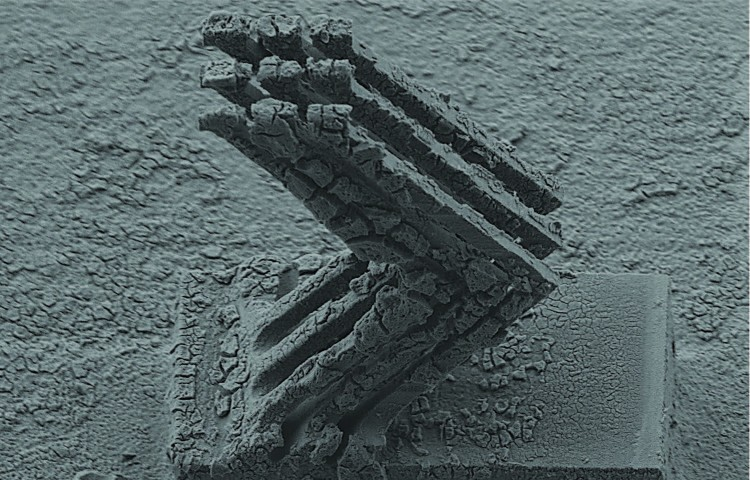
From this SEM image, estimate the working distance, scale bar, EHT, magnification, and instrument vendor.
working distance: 14 mm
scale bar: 100000 nm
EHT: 15 kV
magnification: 0.09 K X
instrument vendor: JEOL